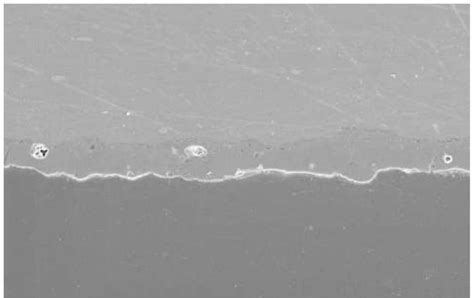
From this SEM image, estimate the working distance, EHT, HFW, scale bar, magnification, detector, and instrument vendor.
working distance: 12.4 mm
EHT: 10 kV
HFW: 254 µm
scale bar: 50000 nm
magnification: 0.5 K X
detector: ETD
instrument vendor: Thermo